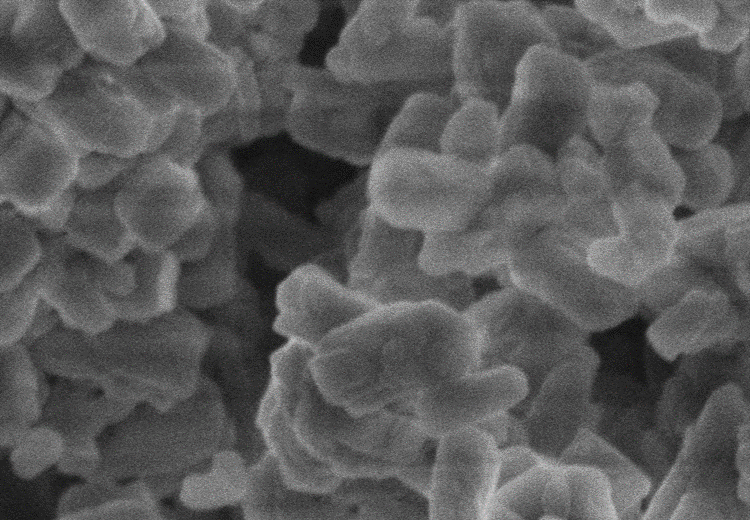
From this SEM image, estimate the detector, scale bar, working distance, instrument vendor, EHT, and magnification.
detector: SE(M)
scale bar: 1000 nm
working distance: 12.1 mm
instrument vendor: Hitachi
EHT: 5 kV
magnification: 30 K X